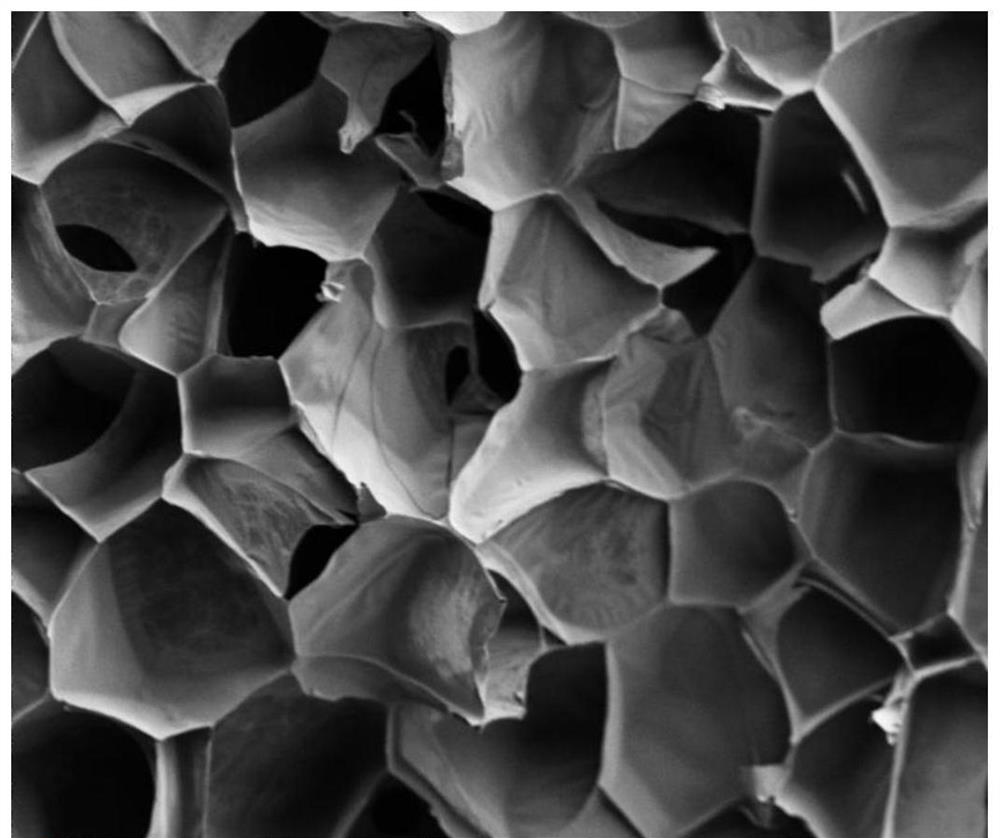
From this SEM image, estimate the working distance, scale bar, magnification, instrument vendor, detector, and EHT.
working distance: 10.2 mm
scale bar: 100000 nm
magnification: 1 K X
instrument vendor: Thermo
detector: ETD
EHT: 2 kV